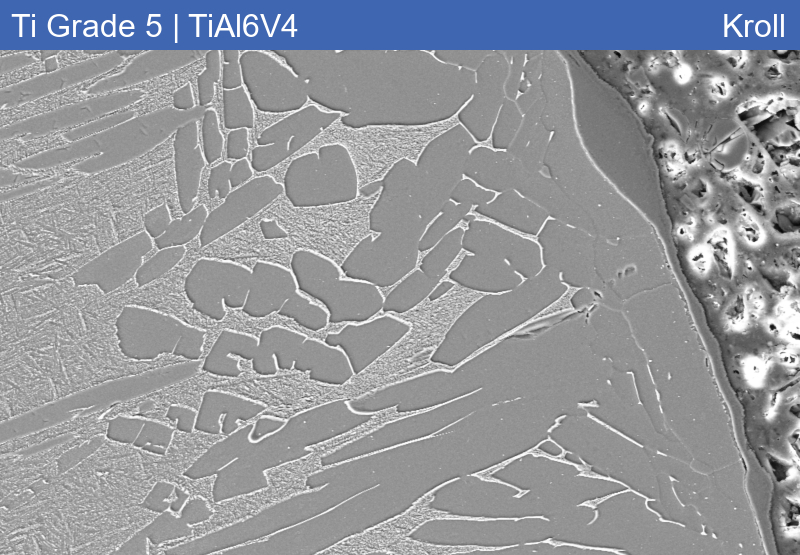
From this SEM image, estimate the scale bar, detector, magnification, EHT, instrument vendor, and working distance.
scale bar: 20000 nm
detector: SE2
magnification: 0.5 K X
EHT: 15 kV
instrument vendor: Zeiss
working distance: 10.1 mm